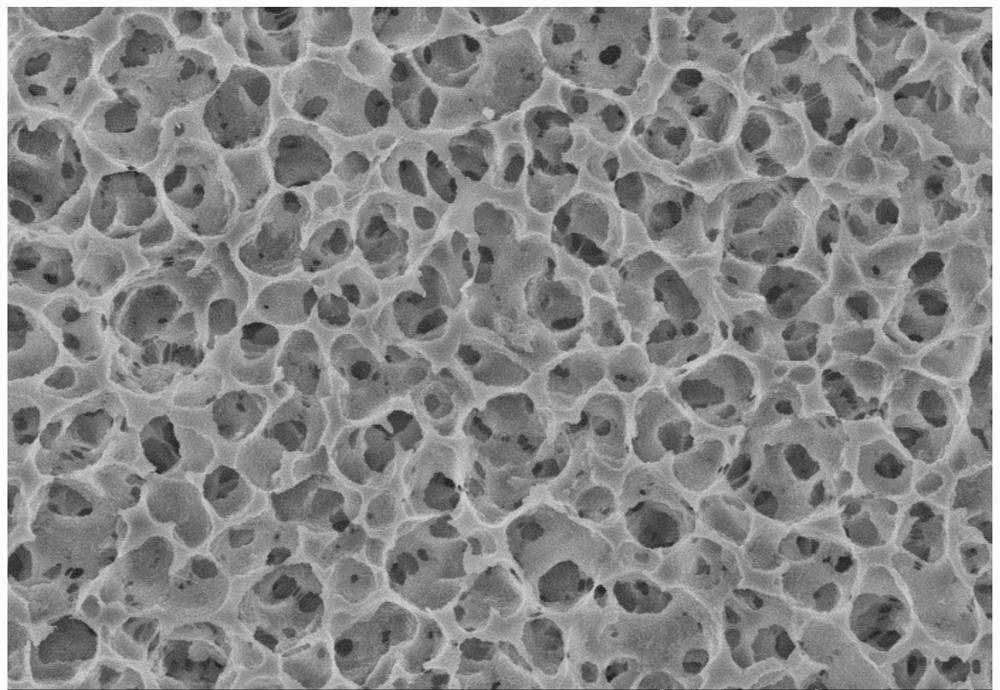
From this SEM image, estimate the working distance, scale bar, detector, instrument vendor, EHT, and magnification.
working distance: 8.7 mm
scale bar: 20000 nm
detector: SE(UL)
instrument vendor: Hitachi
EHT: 10 kV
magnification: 2 K X